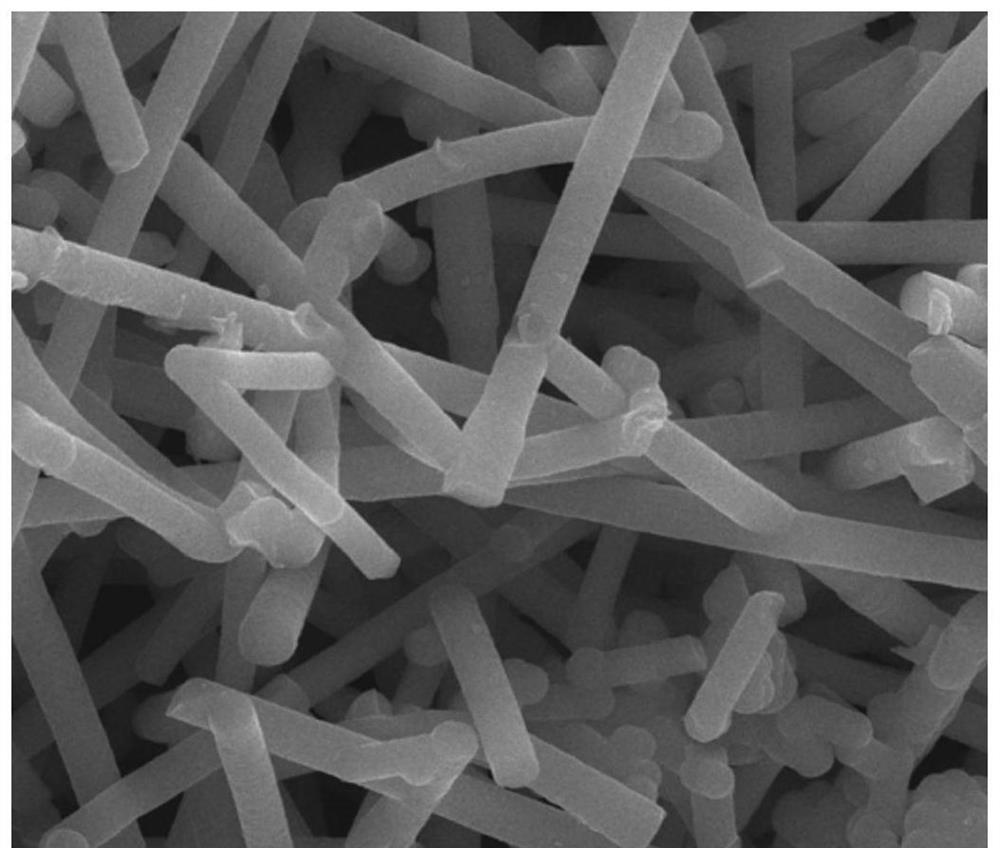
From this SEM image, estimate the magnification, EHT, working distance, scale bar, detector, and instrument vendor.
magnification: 20 K X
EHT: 20 kV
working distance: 9.4 mm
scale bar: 5000 nm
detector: ETD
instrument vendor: FEI